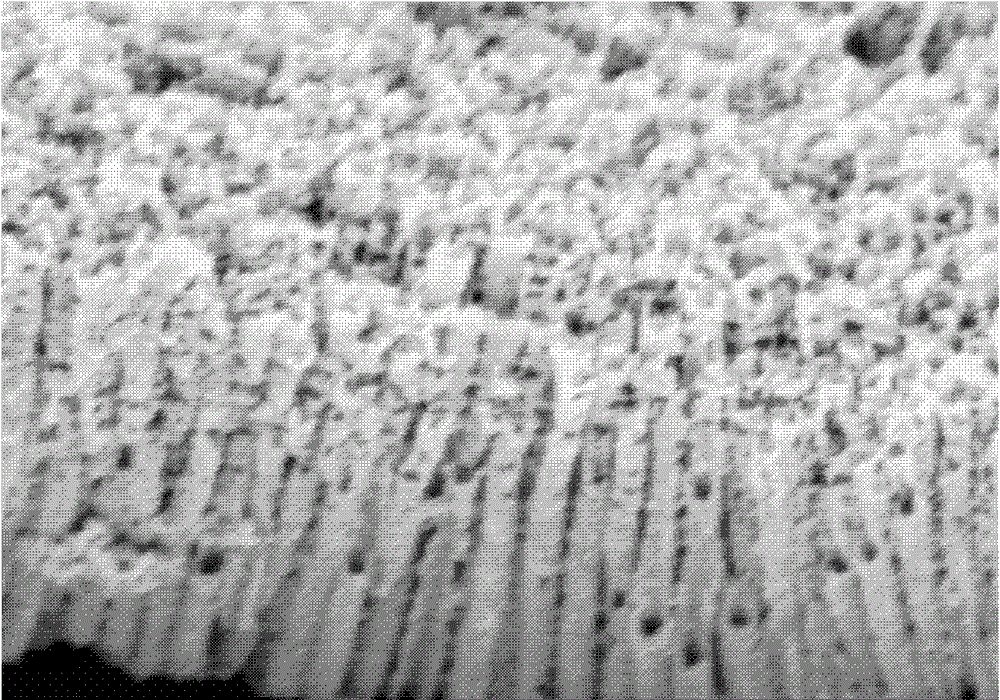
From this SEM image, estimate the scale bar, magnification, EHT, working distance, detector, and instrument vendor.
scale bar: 500 nm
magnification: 80 K X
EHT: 1 kV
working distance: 3.6 mm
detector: SE(U)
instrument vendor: Hitachi